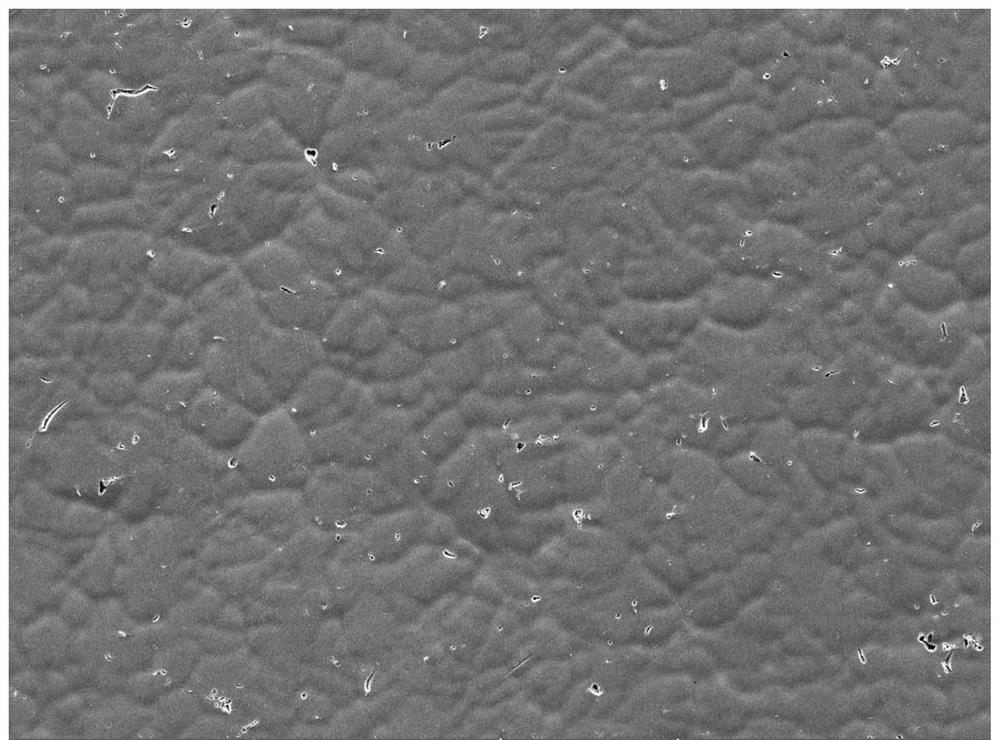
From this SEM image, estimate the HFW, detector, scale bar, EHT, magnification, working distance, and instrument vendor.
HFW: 554 µm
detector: SE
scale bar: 100000 nm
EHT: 20 kV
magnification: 0.5 K X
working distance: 14.4 mm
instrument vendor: Tescan